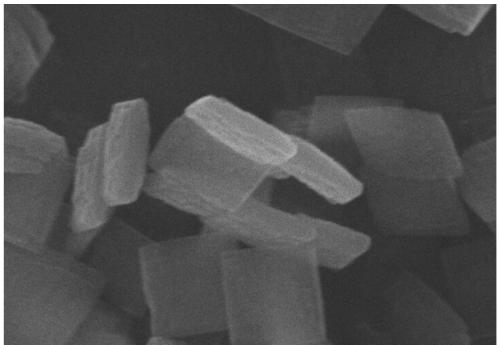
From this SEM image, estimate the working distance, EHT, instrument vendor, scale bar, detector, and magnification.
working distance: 7.9 mm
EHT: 20 kV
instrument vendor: Hitachi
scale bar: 1000 nm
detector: SE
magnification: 40 K X